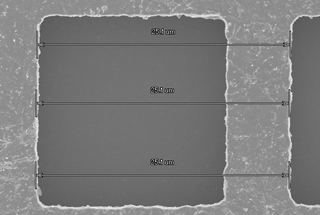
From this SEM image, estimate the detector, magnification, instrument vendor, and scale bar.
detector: SE(UL)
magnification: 4 K X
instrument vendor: Hitachi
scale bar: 10000 nm